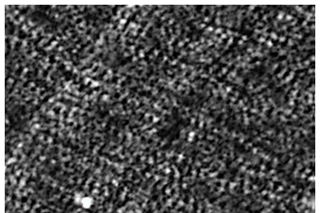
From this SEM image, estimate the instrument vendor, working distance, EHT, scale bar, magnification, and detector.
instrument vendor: JEOL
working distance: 10 mm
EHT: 10 kV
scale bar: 10000 nm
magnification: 2.5 K X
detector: SED